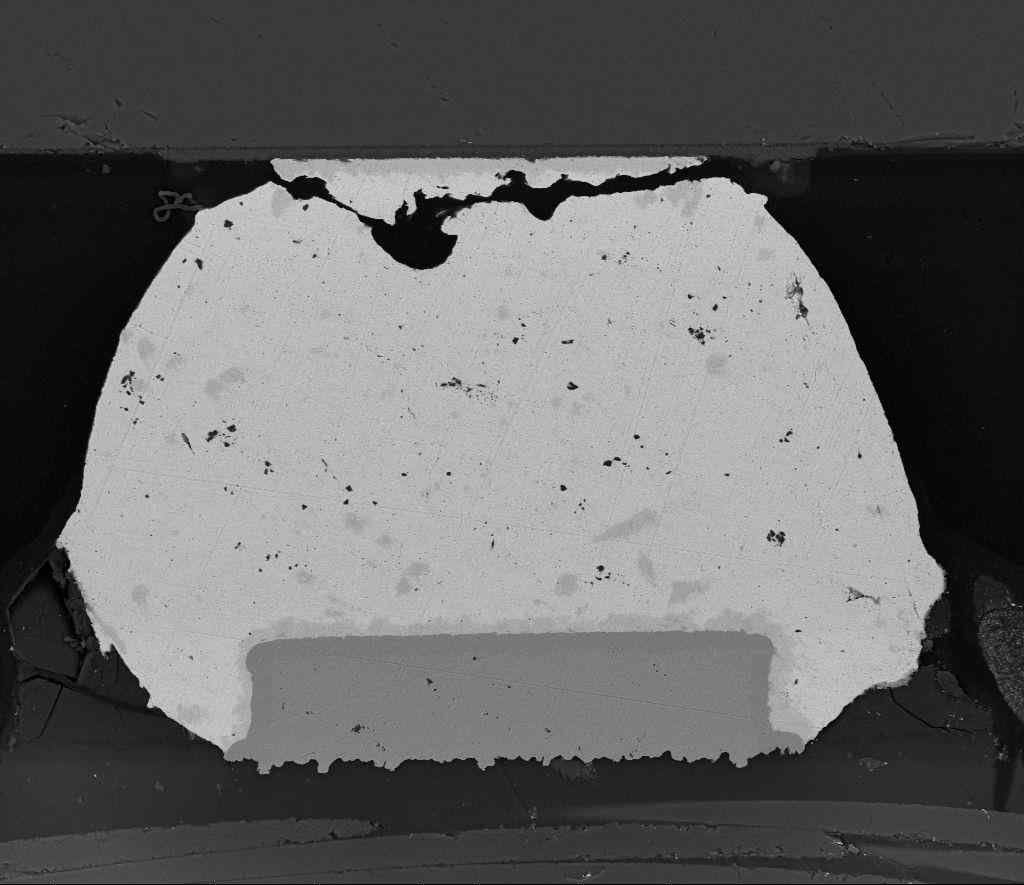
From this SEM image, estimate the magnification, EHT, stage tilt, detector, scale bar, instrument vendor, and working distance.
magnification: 1 K X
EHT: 15 kV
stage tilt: -0°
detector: BSED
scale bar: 100000 nm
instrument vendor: FEI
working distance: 7.1 mm